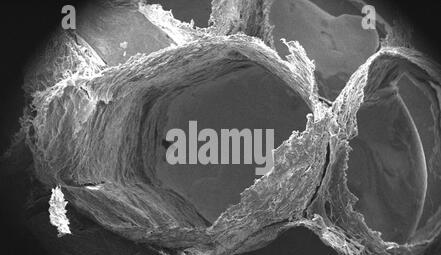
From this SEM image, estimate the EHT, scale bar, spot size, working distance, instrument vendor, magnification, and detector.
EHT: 30 kV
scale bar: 2000000 nm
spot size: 3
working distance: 24.7 mm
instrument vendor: FEI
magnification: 0.012 K X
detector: SE